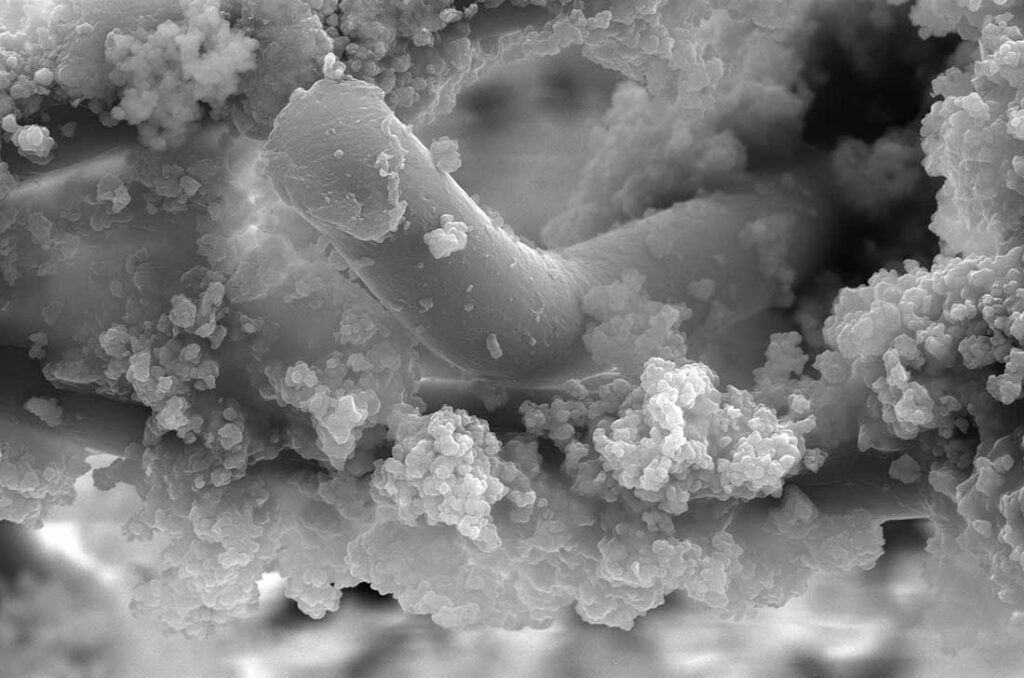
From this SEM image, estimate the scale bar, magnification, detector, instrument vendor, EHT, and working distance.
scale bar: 30000 nm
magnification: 2 K X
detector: ETD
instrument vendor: FEI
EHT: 30 kV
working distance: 11.2 mm